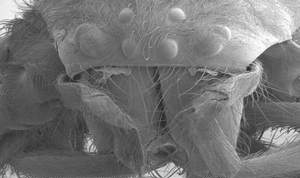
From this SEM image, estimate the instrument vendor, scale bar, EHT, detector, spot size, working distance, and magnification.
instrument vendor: FEI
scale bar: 500000 nm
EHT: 1 kV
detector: SE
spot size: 2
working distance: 14 mm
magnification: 0.07 K X